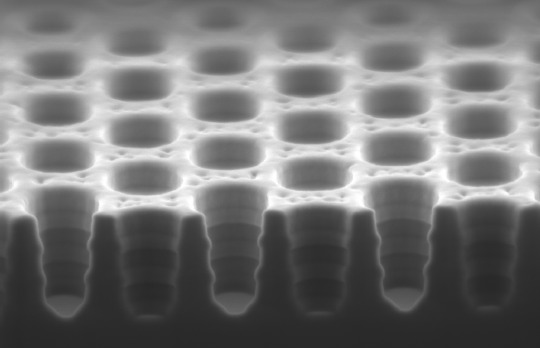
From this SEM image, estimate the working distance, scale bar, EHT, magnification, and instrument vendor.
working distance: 1 mm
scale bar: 200 nm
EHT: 1 kV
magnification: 49.5 K X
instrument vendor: Zeiss